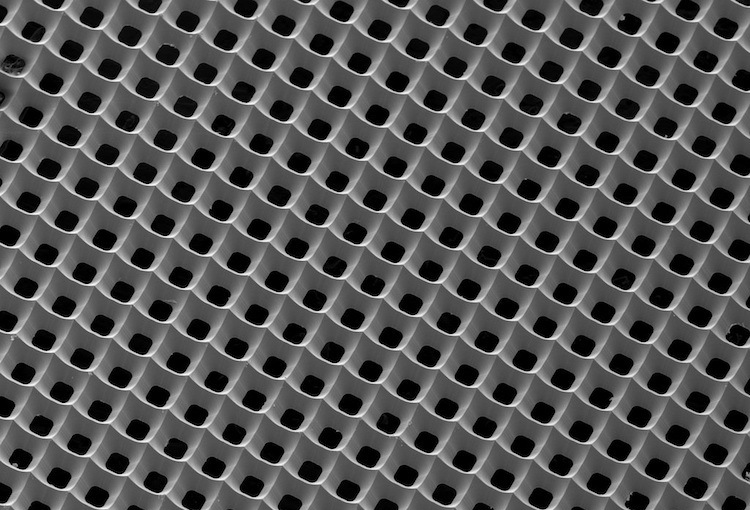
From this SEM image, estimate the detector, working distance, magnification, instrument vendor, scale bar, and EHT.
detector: SEI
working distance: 31 mm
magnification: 0.25 K X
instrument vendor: JEOL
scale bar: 100000 nm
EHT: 10 kV